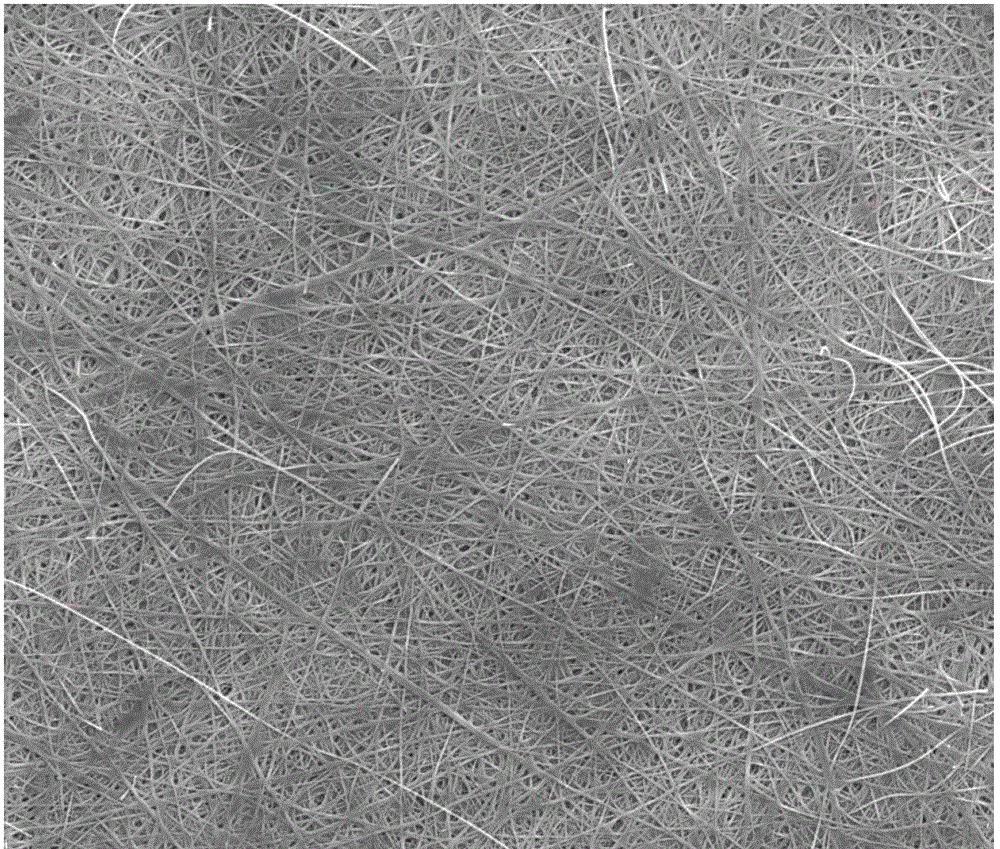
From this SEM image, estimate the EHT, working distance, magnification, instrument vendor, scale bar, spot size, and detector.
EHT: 20 kV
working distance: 17.5 mm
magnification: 5 K X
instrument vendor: FEI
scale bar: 20000 nm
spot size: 4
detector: ETD